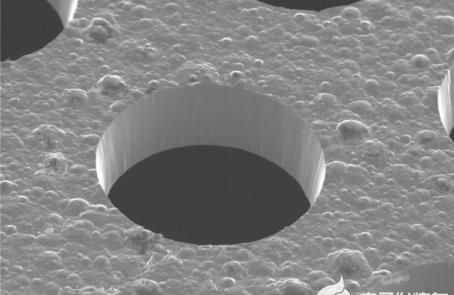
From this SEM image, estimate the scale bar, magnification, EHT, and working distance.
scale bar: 20000 nm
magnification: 0.5 K X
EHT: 20 kV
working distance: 20.5 mm